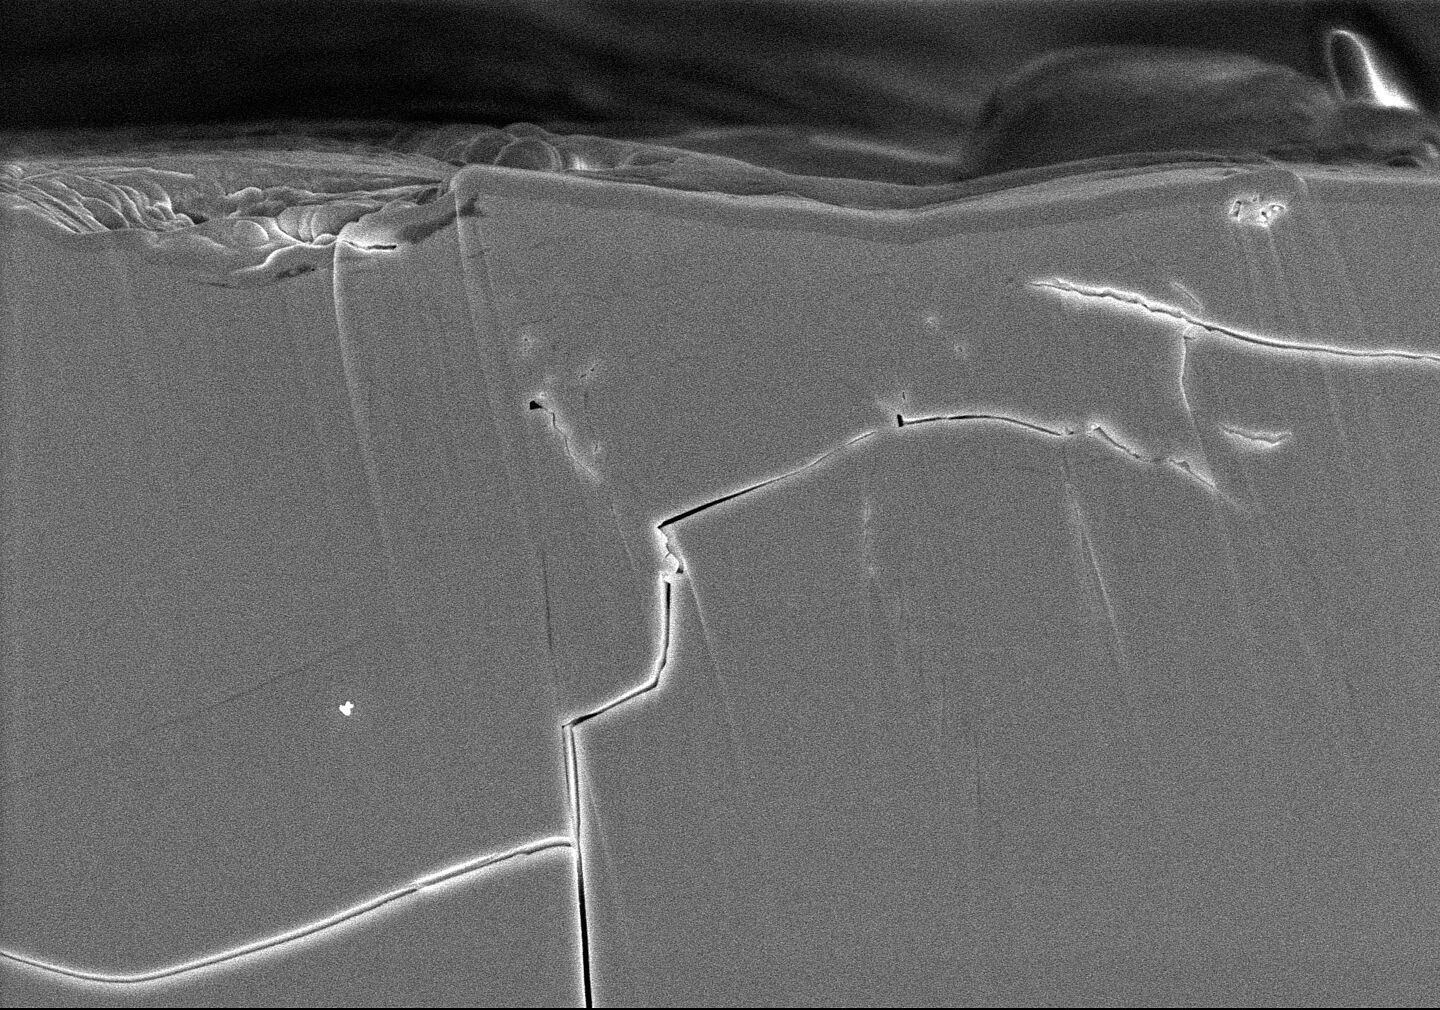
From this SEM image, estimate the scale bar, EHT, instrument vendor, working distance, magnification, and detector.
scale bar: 5000 nm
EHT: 3 kV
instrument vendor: Hitachi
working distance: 16.5 mm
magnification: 10 K X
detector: SE(U)